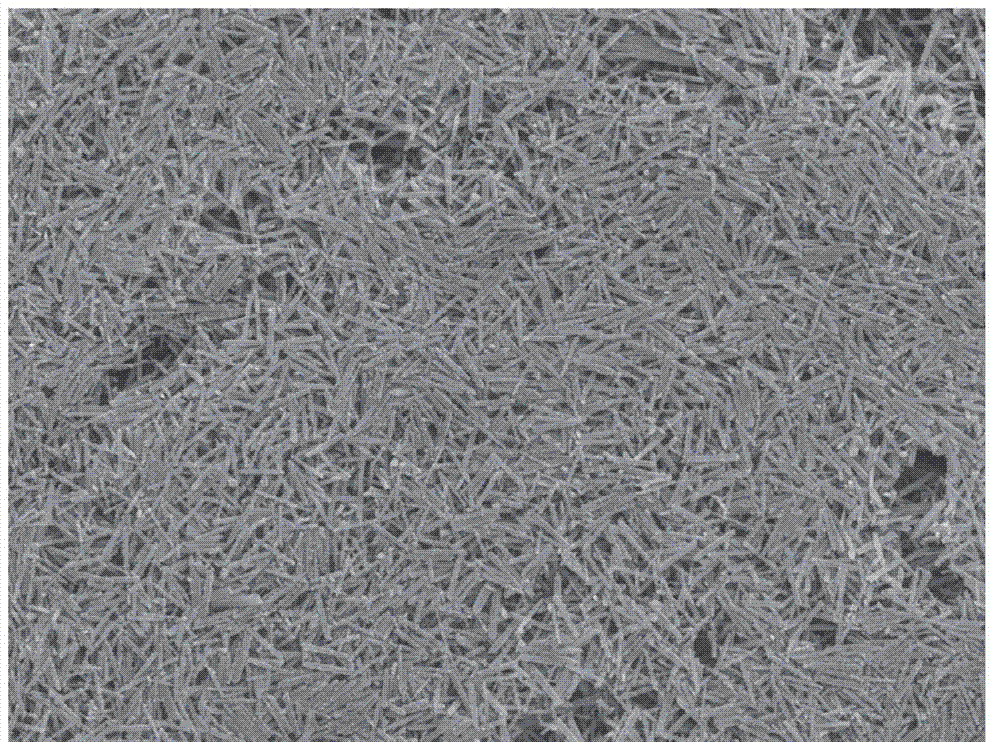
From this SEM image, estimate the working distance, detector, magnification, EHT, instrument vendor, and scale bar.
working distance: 14.5 mm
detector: SEI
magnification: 3 K X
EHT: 15 kV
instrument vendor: JEOL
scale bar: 1000 nm